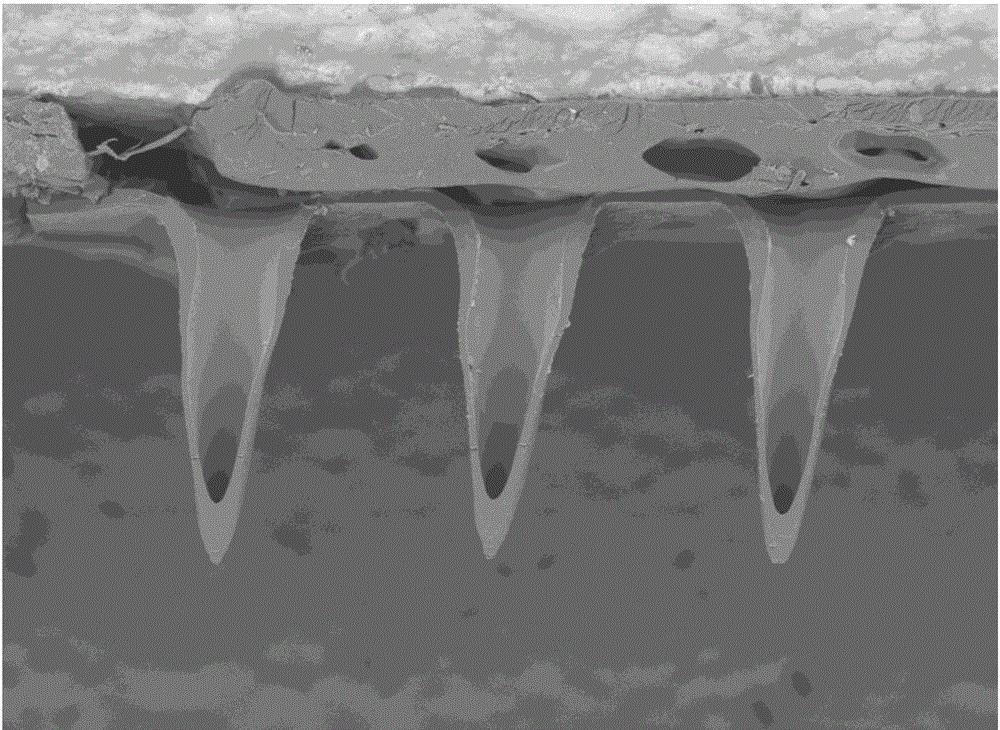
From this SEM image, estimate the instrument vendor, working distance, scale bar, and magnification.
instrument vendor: Hitachi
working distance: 9.4 mm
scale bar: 1000000 nm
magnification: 0.1 K X